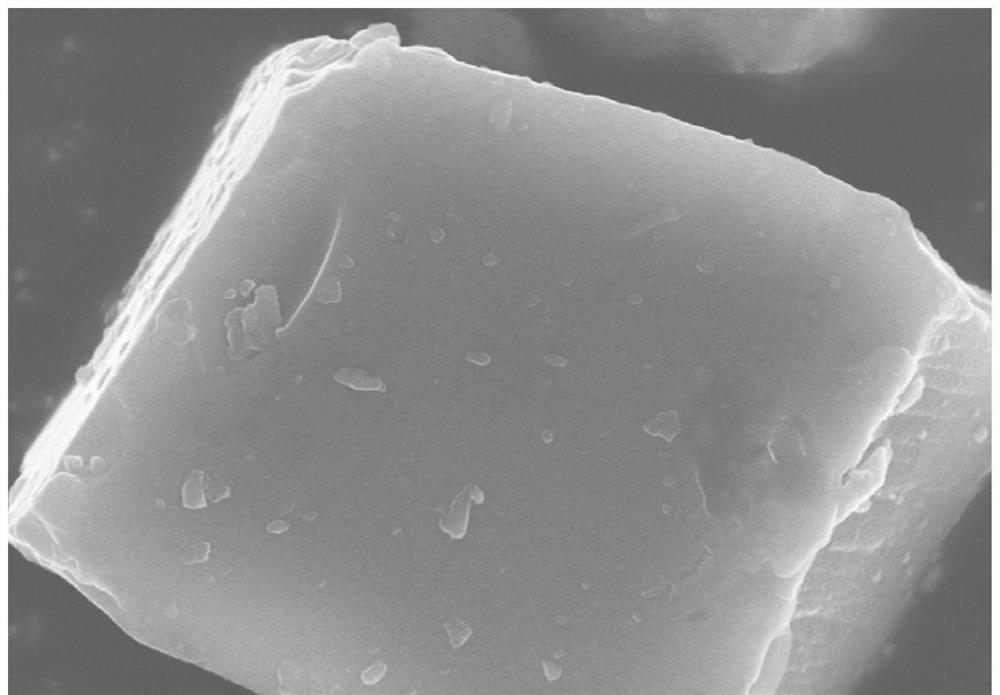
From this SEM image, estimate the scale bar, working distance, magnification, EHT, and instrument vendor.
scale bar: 2000 nm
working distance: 8 mm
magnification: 20 K X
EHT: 10 kV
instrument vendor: Hitachi